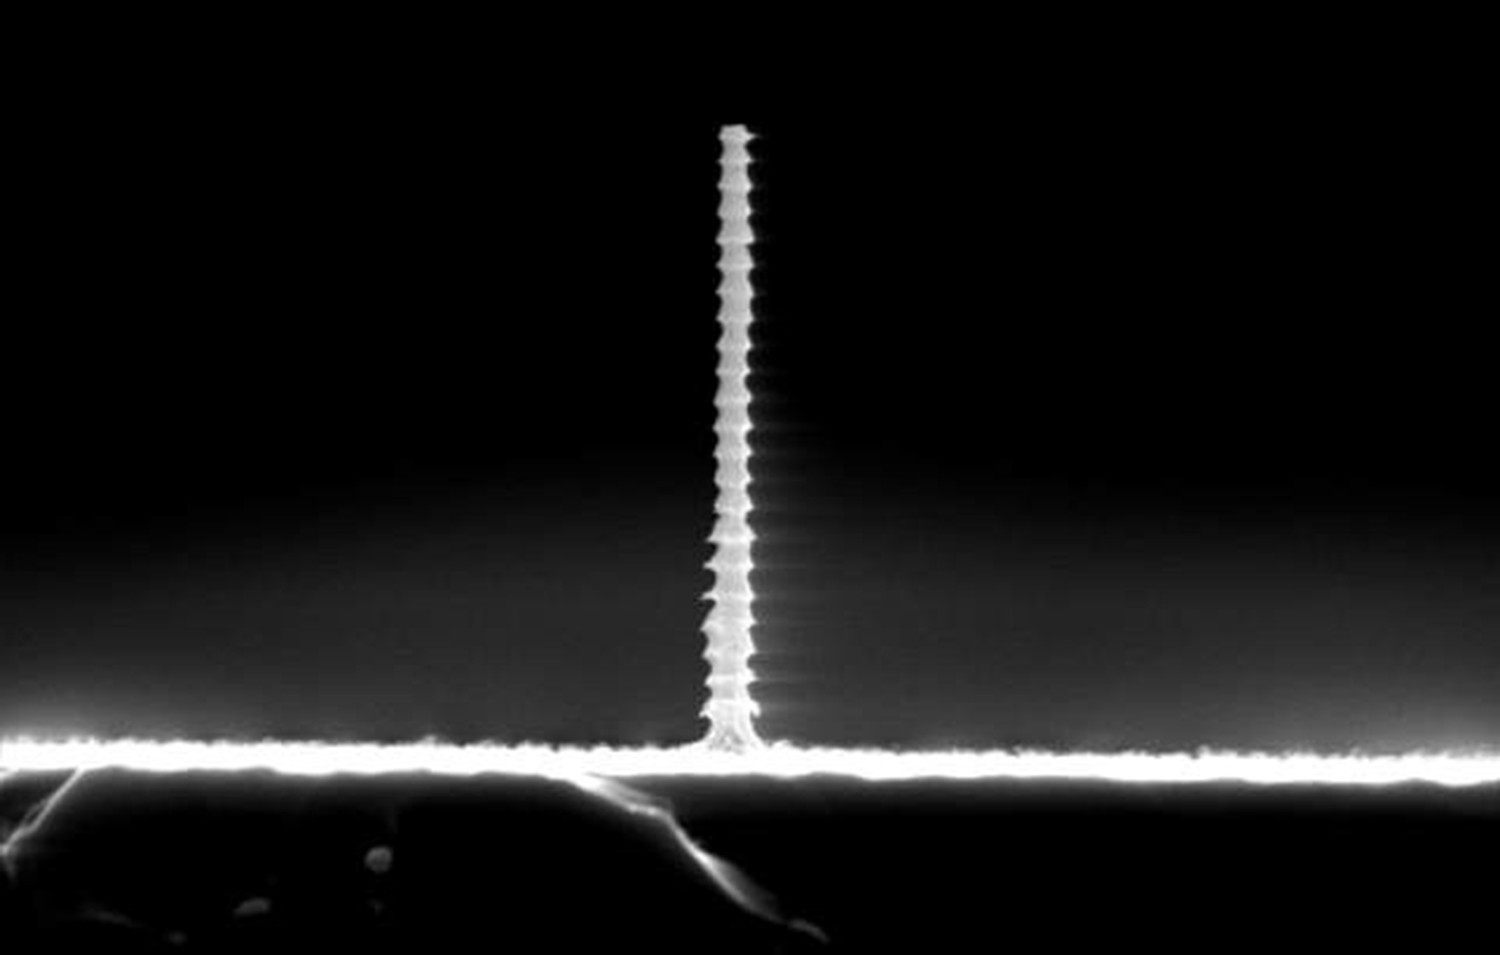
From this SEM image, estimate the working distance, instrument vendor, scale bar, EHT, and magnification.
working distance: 7 mm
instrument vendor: JEOL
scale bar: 5000 nm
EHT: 15 kV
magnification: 5 K X